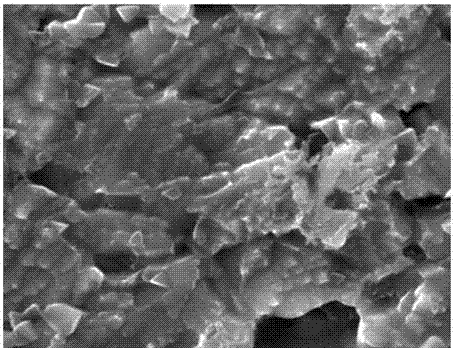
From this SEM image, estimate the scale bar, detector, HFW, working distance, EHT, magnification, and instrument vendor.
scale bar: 2000 nm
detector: SE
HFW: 10.4 µm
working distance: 15.48 mm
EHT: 30 kV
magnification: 20 K X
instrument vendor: Tescan